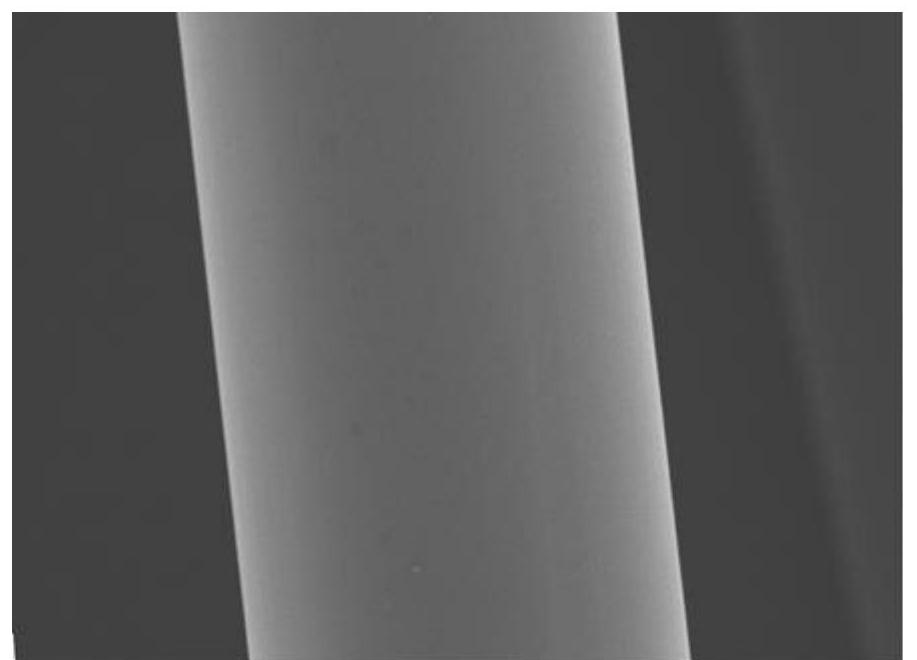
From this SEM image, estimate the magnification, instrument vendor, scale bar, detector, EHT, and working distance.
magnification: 5 K X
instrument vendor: JEOL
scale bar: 1000 nm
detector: SEI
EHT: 10 kV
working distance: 9 mm